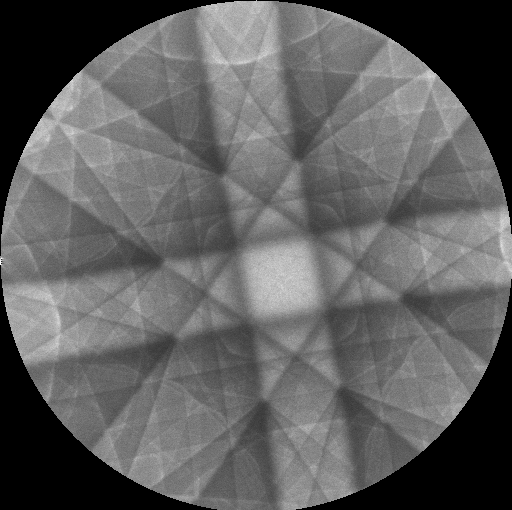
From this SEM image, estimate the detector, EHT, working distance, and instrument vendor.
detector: BSE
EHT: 20 kV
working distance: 11.85 mm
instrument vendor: Tescan